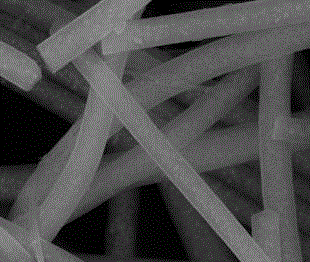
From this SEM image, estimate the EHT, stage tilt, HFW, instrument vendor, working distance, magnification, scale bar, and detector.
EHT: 20 kV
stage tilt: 0°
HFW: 5.97 µm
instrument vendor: FEI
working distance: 12.3 mm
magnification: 50 K X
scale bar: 2000 nm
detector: ETD